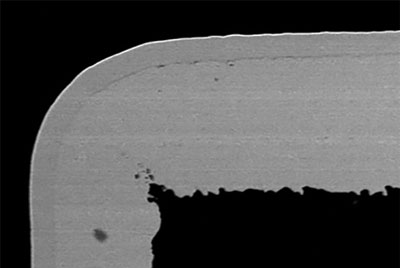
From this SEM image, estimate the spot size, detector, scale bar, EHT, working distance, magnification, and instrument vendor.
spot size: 60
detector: BEC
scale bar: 10000 nm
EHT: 15 kV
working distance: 15 mm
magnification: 1 K X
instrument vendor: JEOL